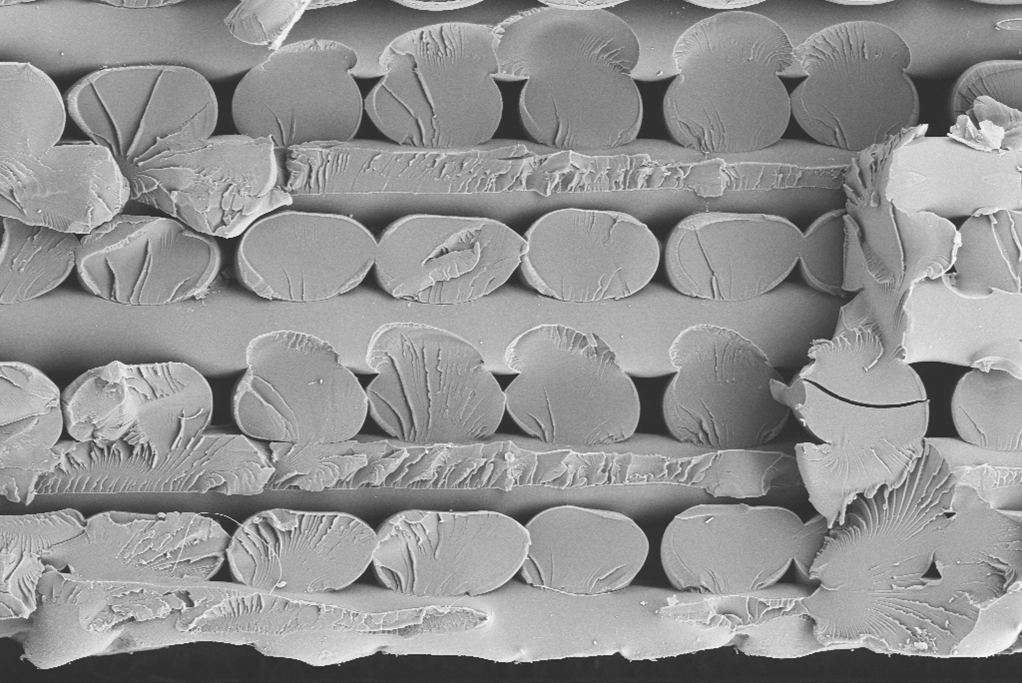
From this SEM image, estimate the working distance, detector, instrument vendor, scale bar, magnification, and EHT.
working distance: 8 mm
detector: RBSD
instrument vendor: Zeiss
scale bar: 100000 nm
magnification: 0.15 K X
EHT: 20 kV